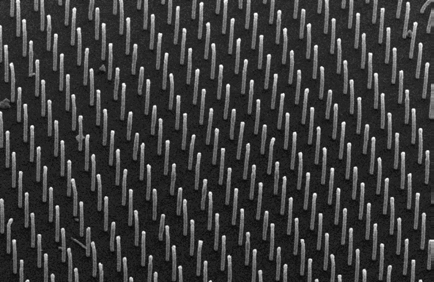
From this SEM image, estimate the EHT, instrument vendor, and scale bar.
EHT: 15 kV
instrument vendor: JEOL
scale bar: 200000 nm